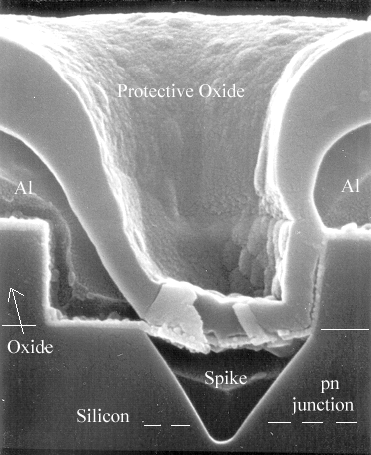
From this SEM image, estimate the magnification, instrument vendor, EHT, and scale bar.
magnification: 30 K X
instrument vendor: JEOL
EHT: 25 kV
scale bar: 1000 nm